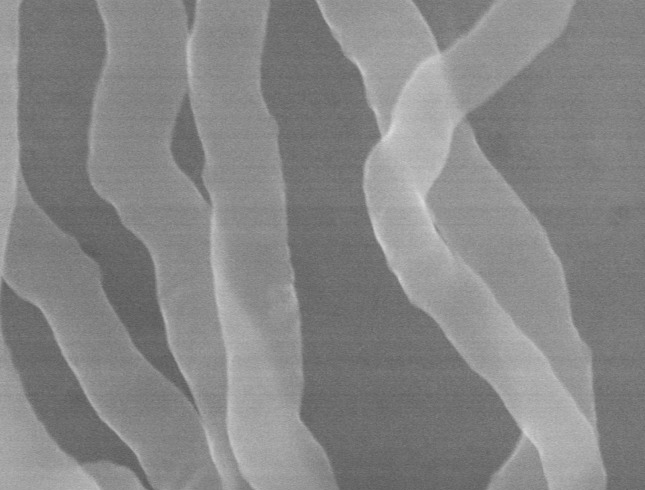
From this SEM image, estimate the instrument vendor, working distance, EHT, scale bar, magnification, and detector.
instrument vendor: Hitachi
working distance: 8.5 mm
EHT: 30 kV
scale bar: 500 nm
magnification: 120 K X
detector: SE(UL)